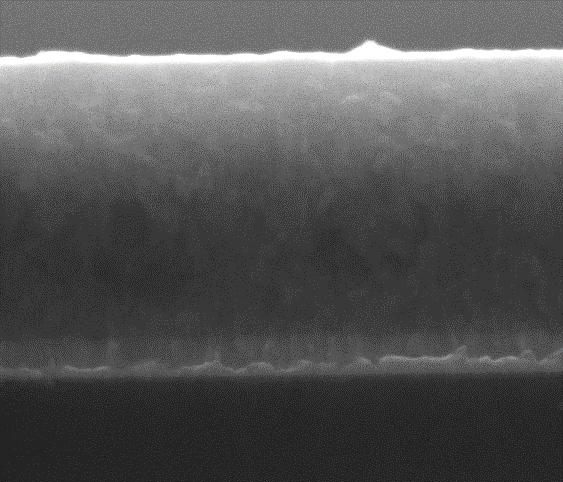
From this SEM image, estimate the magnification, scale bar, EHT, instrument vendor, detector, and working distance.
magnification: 100 K X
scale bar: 1000 nm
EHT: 15 kV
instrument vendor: FEI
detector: ETD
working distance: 10.8 mm